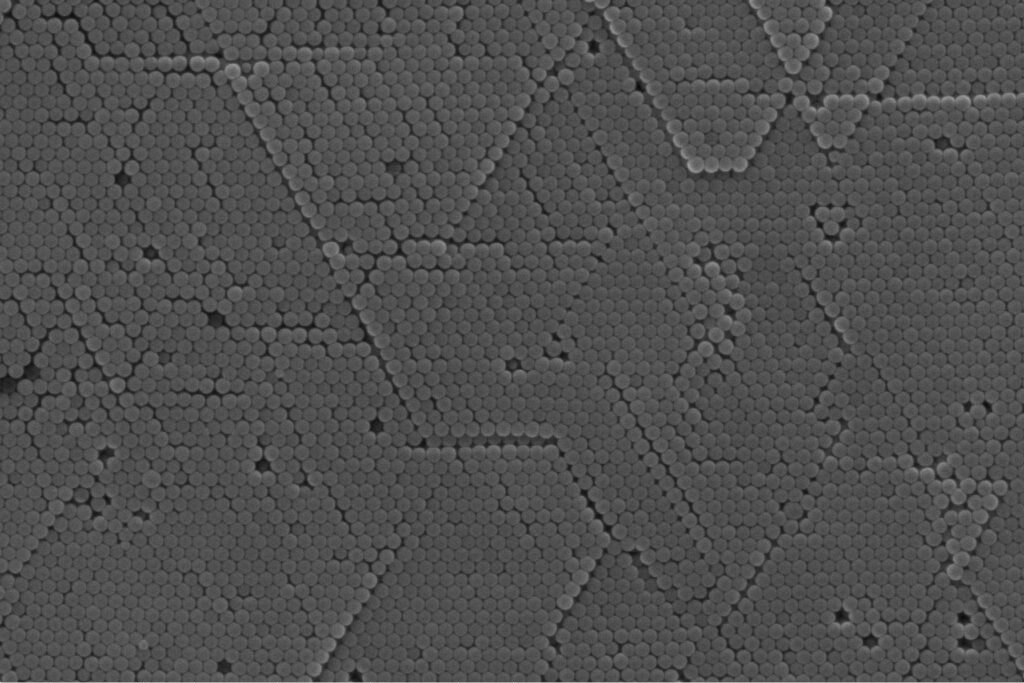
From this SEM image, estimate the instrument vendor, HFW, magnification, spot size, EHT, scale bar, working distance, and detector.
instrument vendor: FEI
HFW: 8.29 µm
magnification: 50 K X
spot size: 4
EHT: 20 kV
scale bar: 3000 nm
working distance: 9.9 mm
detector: ETD